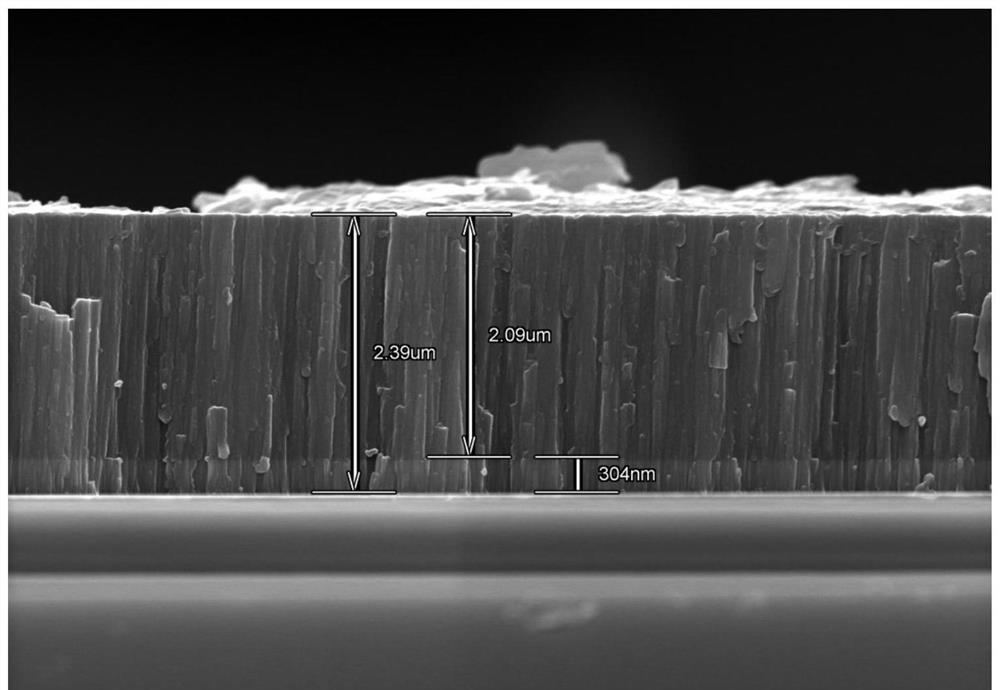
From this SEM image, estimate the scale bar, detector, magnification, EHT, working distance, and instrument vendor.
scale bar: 3000 nm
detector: SE(U)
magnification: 15 K X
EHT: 15 kV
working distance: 11.2 mm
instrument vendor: Hitachi